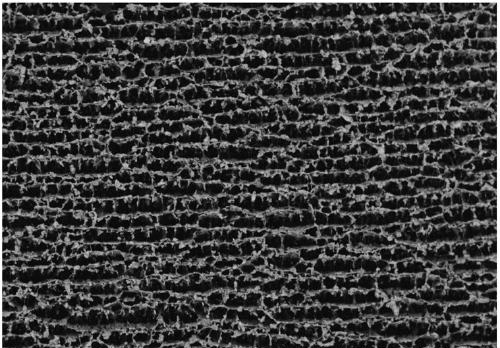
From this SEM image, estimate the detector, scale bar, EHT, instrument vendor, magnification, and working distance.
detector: BSE-COMP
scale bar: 400000 nm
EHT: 5 kV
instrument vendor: Hitachi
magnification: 0.13 K X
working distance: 8.2 mm